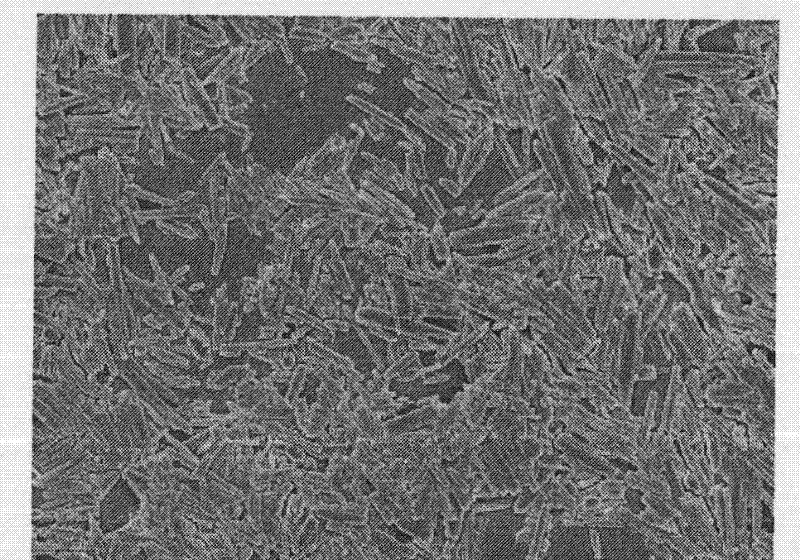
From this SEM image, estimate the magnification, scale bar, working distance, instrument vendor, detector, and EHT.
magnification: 3.5 K X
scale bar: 1000 nm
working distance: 6.2 mm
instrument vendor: JEOL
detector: SEI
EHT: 5 kV